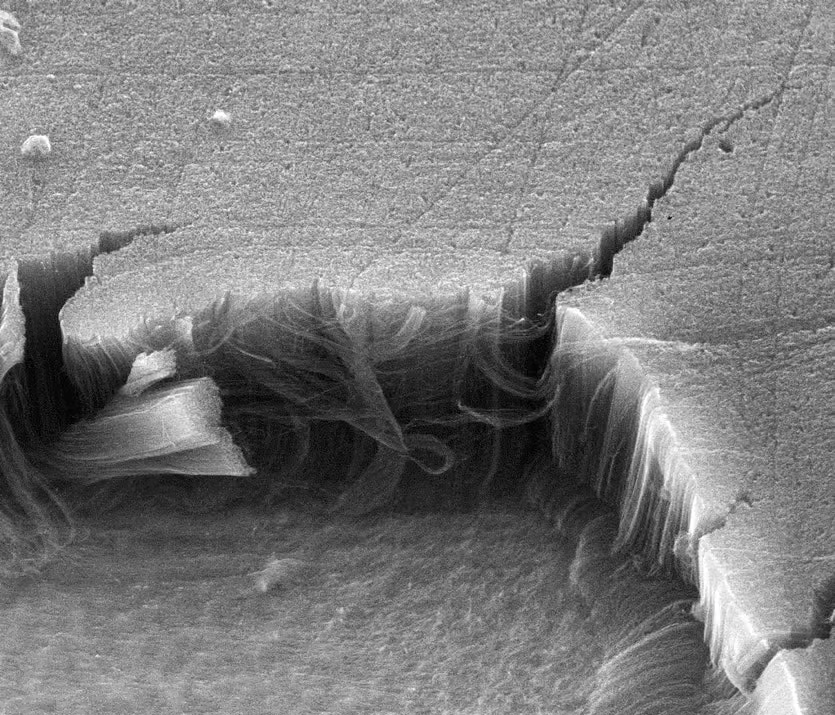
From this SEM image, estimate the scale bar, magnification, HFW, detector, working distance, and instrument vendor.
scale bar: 20000 nm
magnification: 4.294 K X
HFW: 62.98 µm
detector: ETD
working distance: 11.8 mm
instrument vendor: FEI